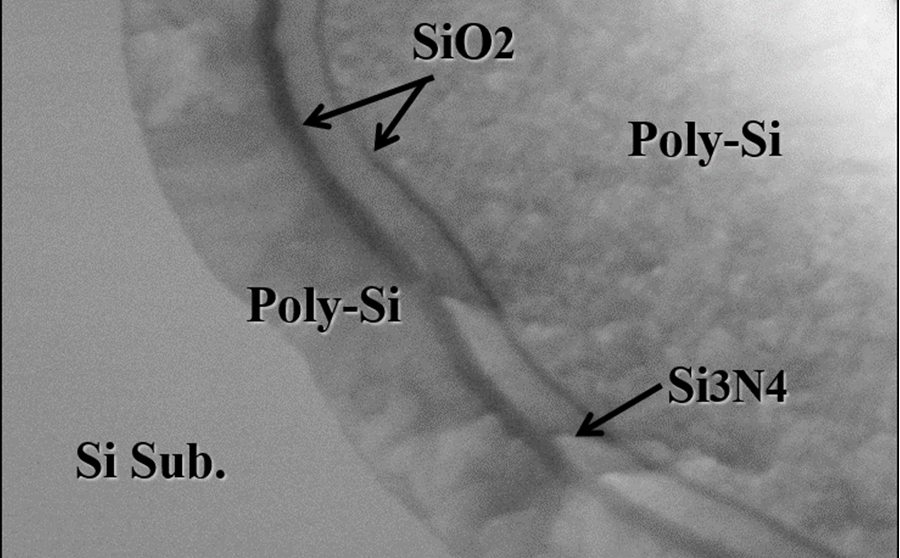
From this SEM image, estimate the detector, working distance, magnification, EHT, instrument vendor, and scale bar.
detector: SE(U,LA20)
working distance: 2 mm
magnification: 150 K X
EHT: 5 kV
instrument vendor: Hitachi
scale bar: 300 nm